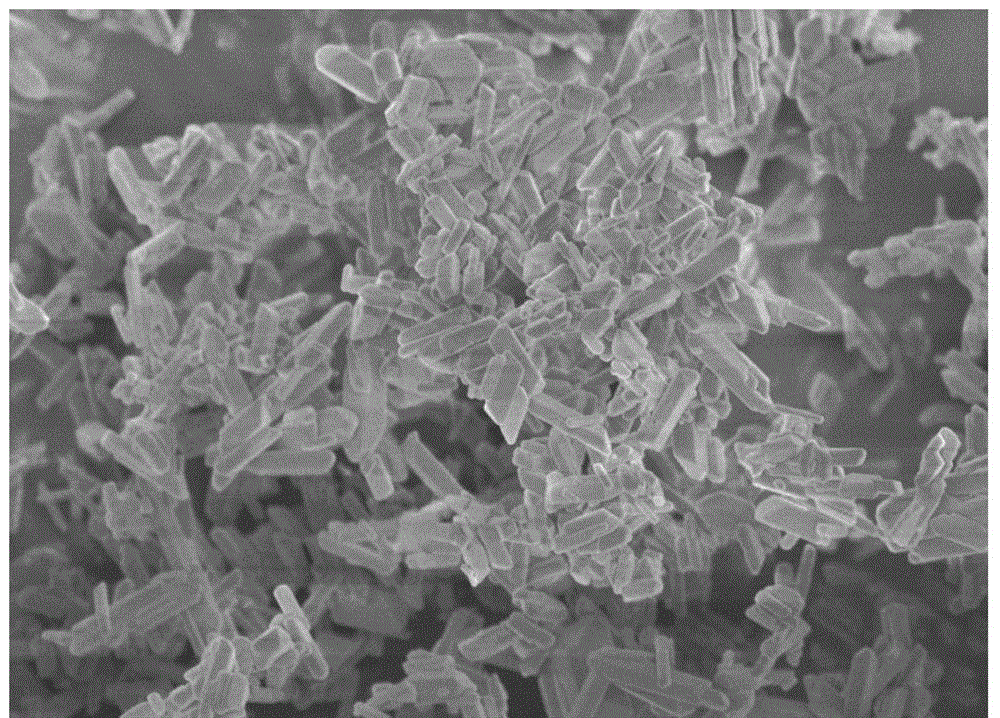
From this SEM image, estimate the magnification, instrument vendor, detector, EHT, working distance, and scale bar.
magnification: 10 K X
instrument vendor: Hitachi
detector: SE(M)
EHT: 3 kV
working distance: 9 mm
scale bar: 5000 nm